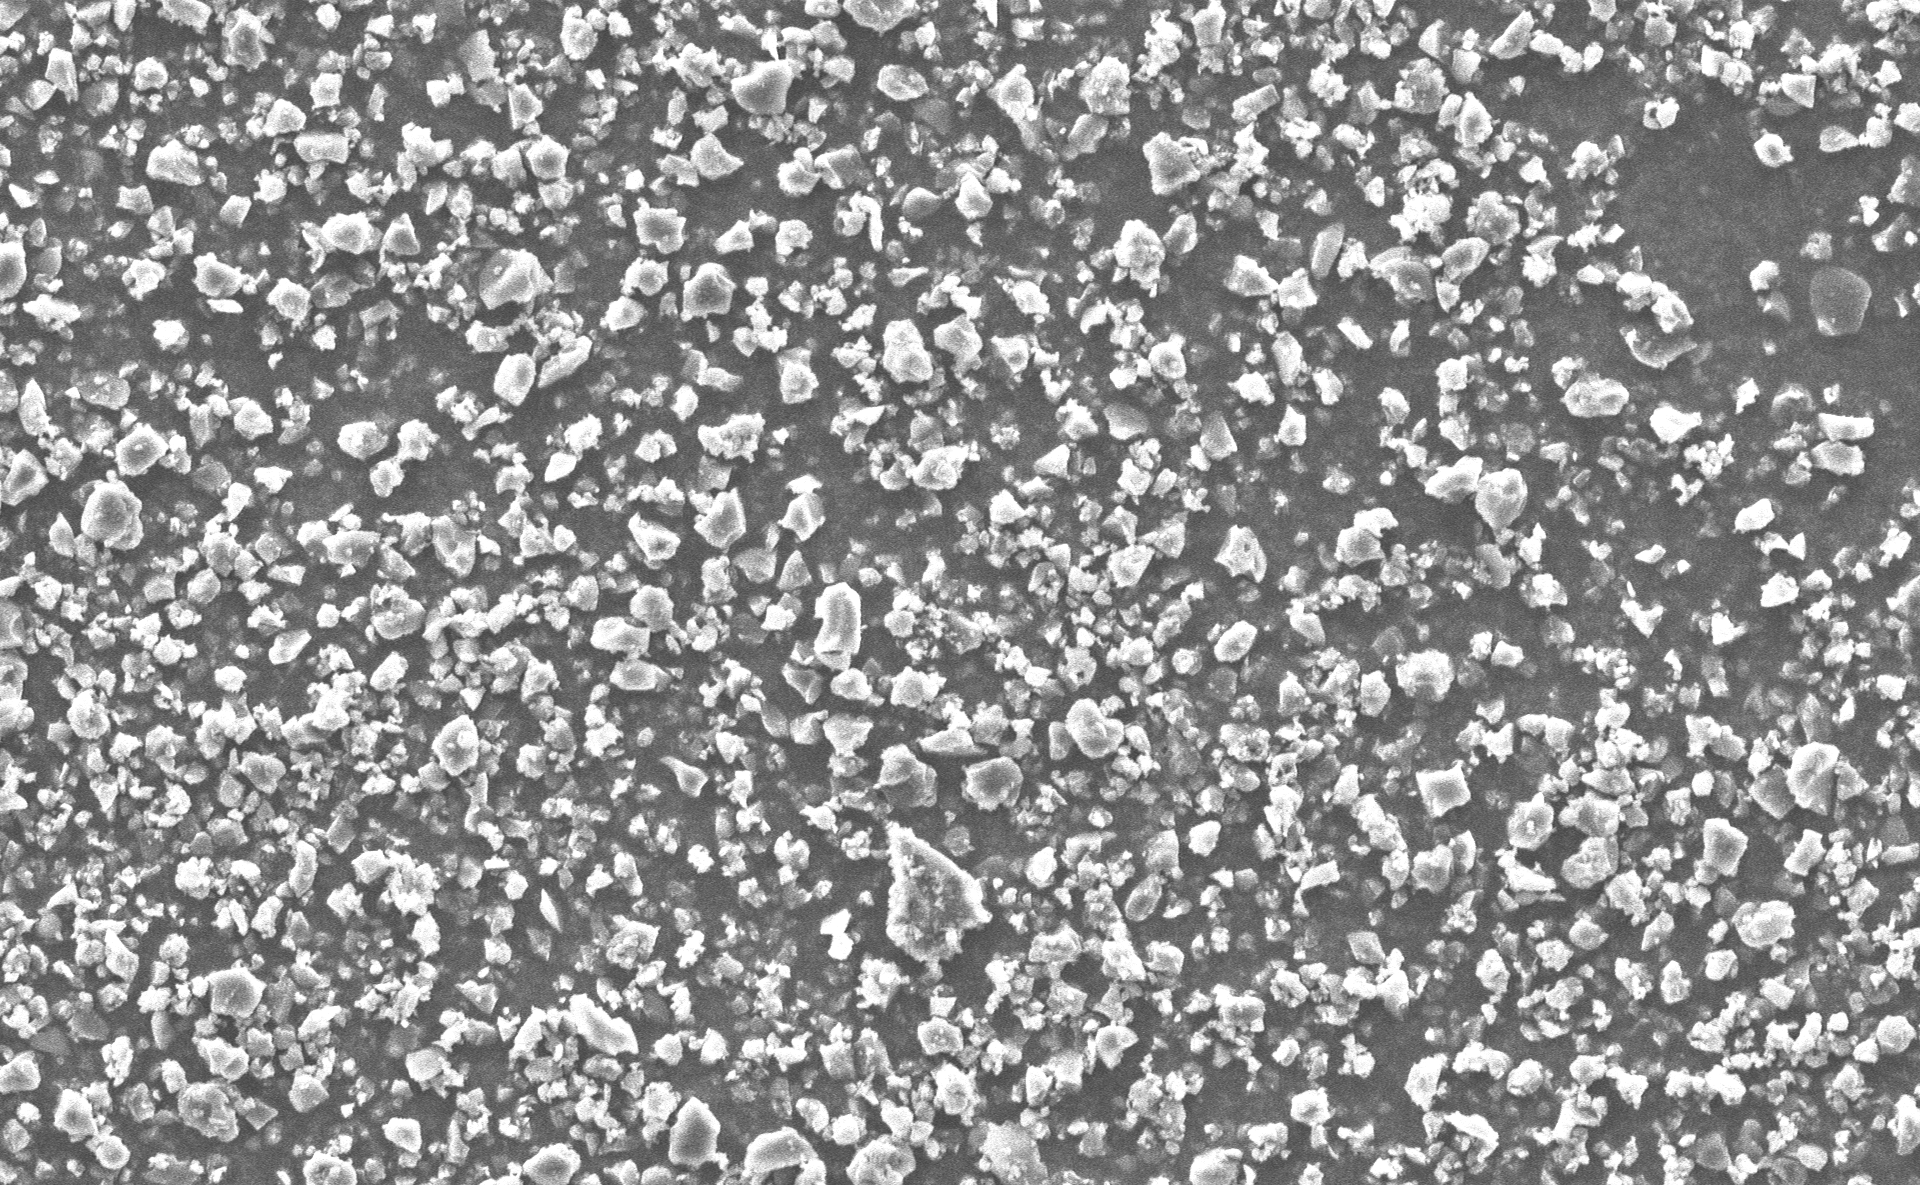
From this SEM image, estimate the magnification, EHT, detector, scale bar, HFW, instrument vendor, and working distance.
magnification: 3 K X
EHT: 10 kV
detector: SED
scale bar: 50000 nm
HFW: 173 µm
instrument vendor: other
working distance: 6.032 mm